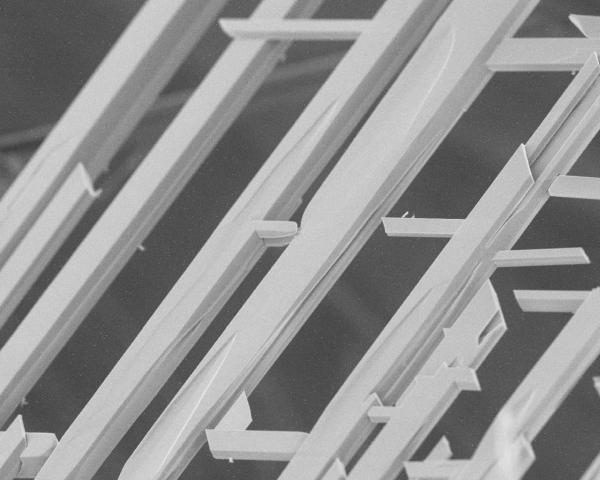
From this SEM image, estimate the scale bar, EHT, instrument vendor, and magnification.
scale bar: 100000 nm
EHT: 10 kV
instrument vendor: JEOL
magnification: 0.3 K X